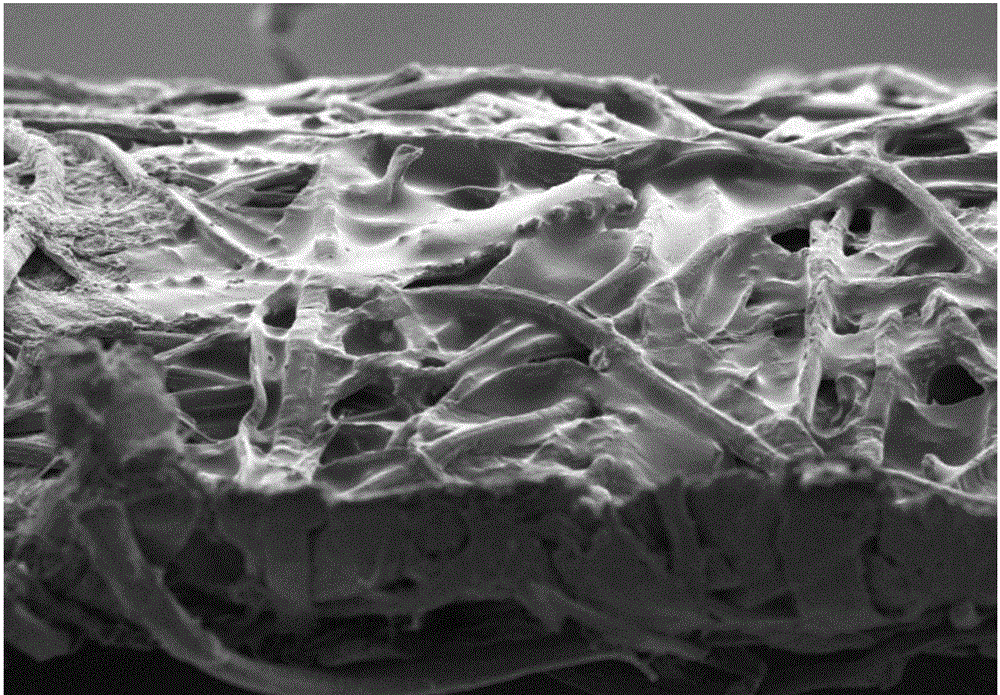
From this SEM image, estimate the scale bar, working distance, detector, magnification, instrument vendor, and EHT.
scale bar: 20000 nm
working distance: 8.8 mm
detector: SE2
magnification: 0.4 K X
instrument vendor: Zeiss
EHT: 5 kV